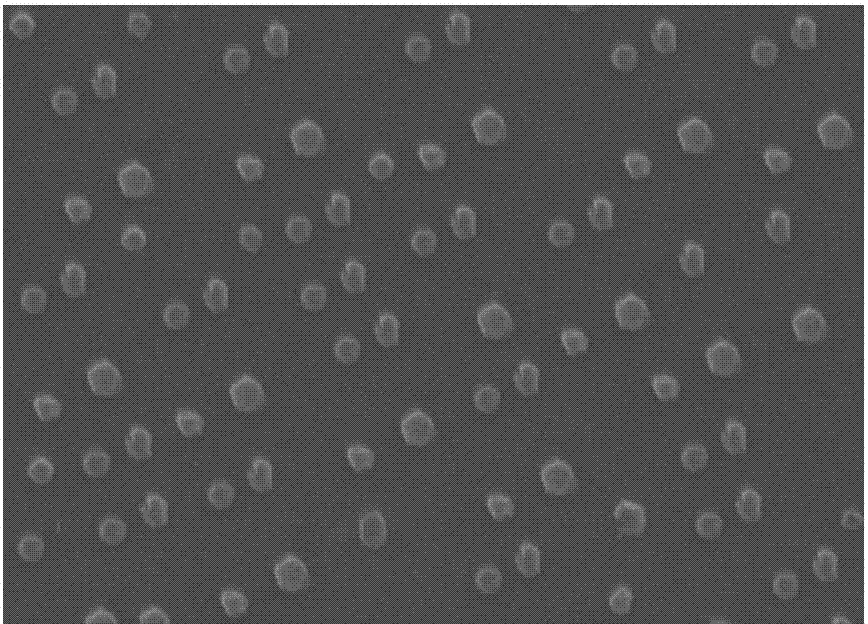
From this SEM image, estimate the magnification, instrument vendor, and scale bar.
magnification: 10 K X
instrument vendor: JEOL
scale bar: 1000 nm